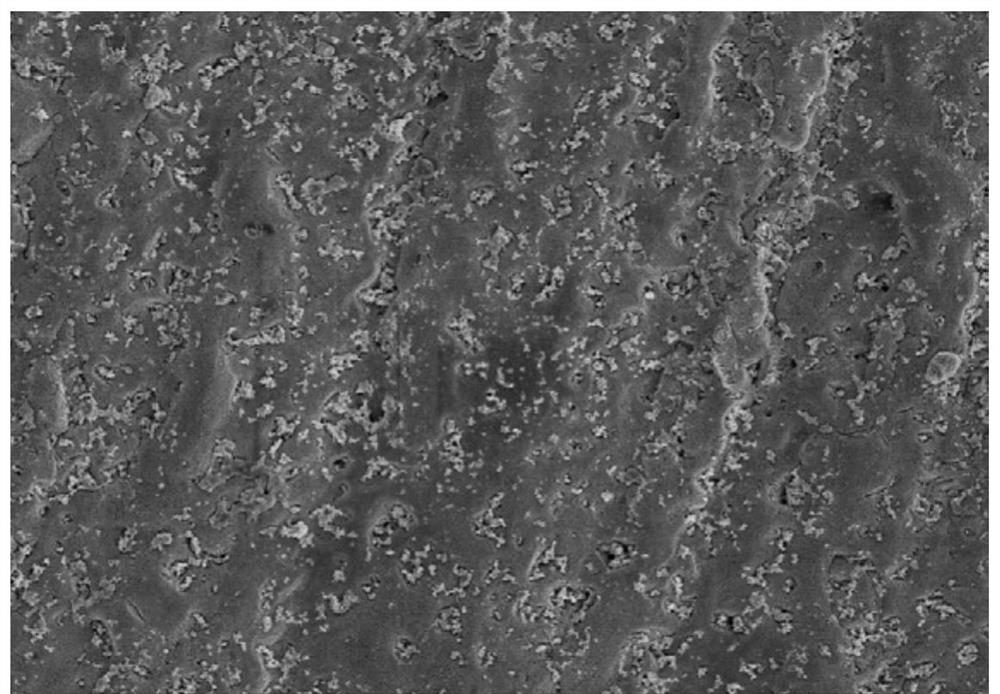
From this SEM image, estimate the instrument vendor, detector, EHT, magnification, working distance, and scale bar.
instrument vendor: Hitachi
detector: SE(M)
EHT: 5 kV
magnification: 2 K X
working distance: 10.7 mm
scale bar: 20000 nm